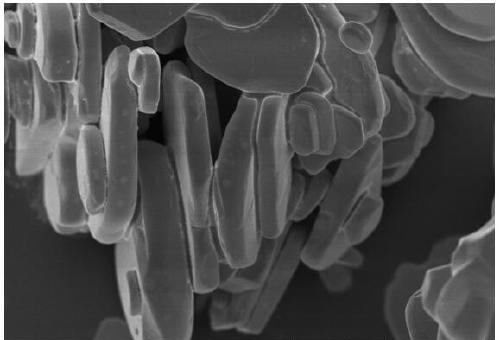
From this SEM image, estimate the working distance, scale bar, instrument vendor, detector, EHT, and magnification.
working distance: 5.4 mm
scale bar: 5000 nm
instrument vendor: Hitachi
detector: SE(UL)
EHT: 3 kV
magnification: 10 K X